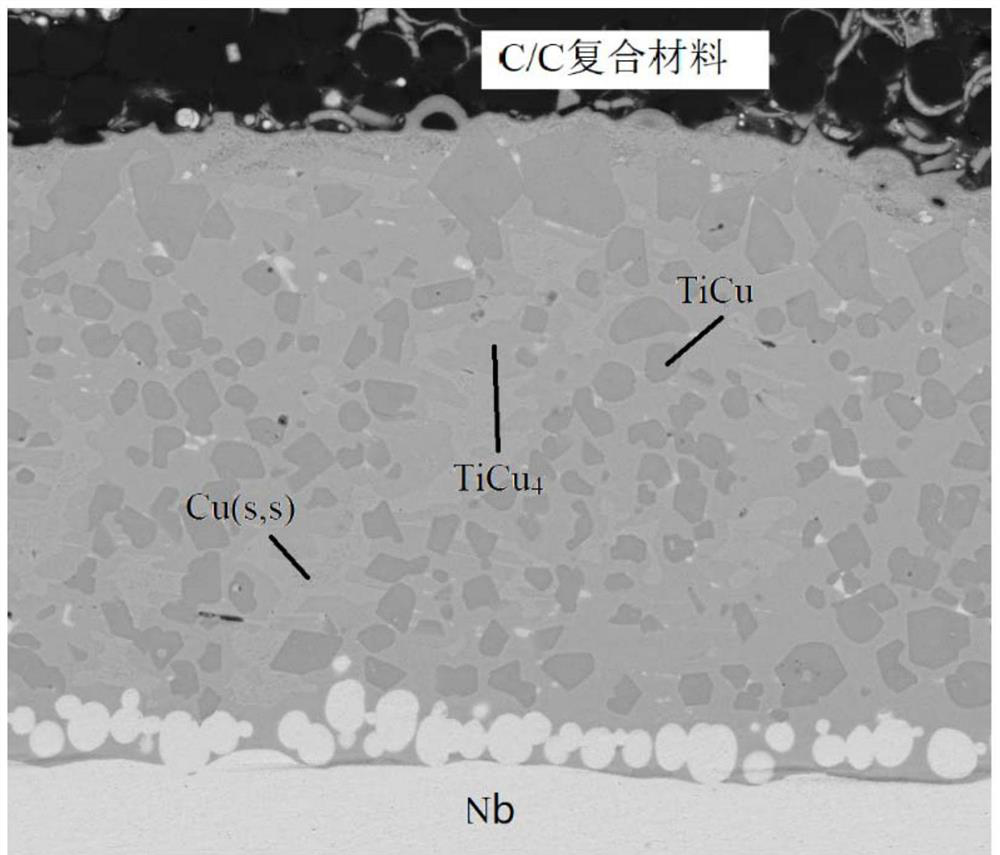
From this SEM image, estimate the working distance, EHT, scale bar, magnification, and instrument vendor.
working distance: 5.9 mm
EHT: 20 kV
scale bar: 30000 nm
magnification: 2.5 K X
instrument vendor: FEI